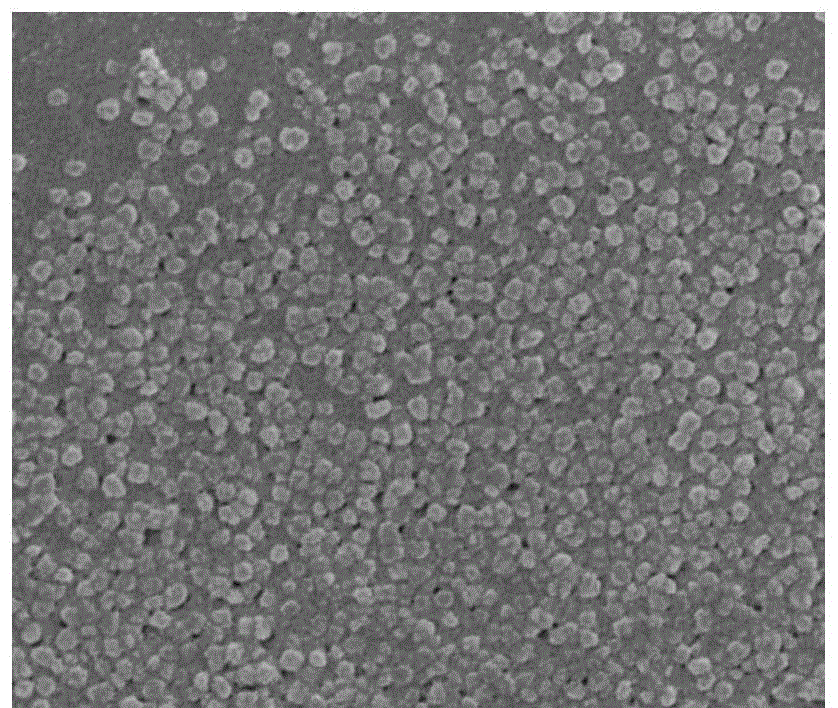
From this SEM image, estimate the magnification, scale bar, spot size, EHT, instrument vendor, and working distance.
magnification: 80 K X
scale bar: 1000 nm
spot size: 3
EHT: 3 kV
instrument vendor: FEI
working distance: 5.1 mm